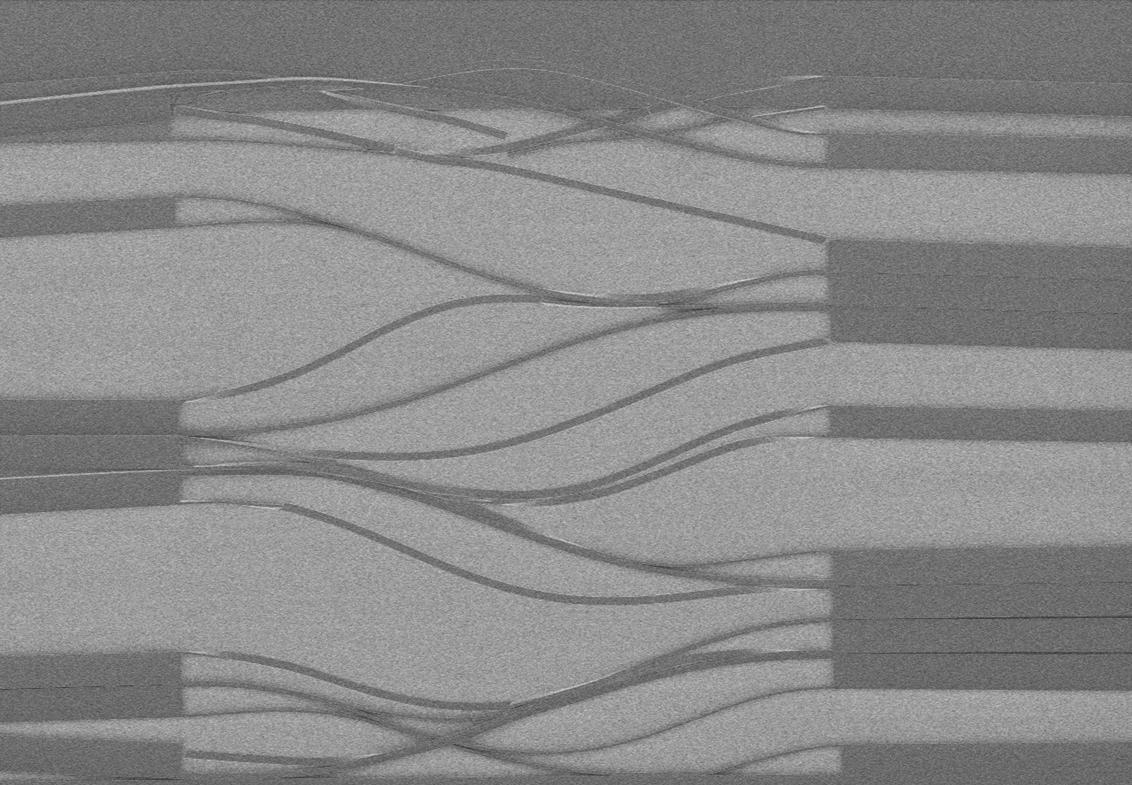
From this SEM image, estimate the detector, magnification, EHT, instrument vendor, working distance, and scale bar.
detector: SE(M)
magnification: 1.5 K X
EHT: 0.7 kV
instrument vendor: Hitachi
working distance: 8.4 mm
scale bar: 30000 nm